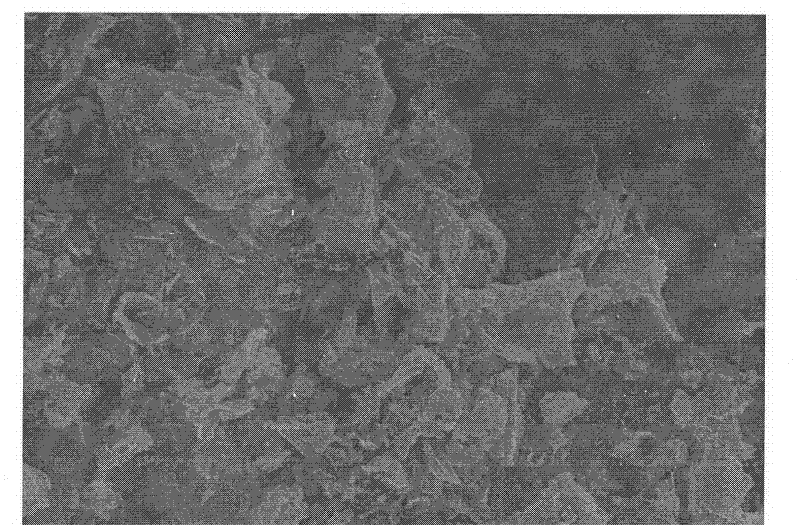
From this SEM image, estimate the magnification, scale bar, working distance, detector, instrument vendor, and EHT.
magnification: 4 K X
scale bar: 10000 nm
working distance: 10.9 mm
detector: SE(M)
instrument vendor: Hitachi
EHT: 10 kV